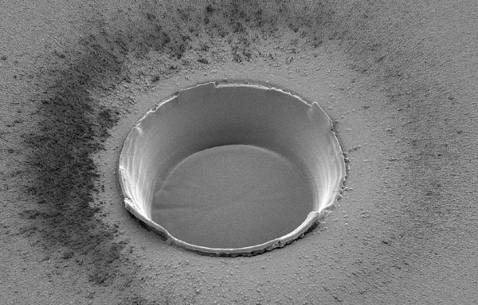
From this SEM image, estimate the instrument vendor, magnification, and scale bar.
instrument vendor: FEI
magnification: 0.466 K X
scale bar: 20000 nm